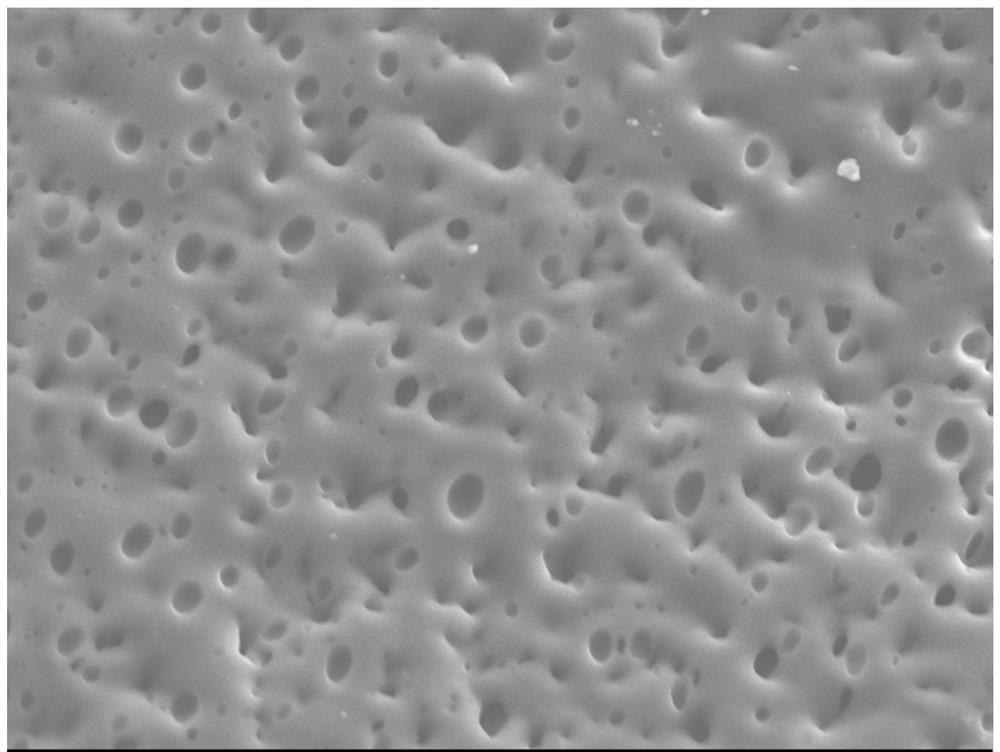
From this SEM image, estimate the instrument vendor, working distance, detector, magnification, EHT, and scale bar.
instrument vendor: JEOL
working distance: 14.8 mm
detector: LEI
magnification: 2 K X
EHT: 10 kV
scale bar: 10000 nm